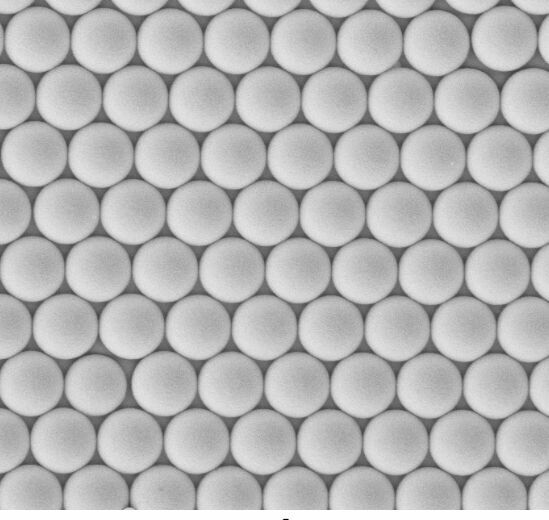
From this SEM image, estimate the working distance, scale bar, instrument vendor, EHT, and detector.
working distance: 7 mm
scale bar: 2000 nm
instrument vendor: Zeiss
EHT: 5 kV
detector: MPSE